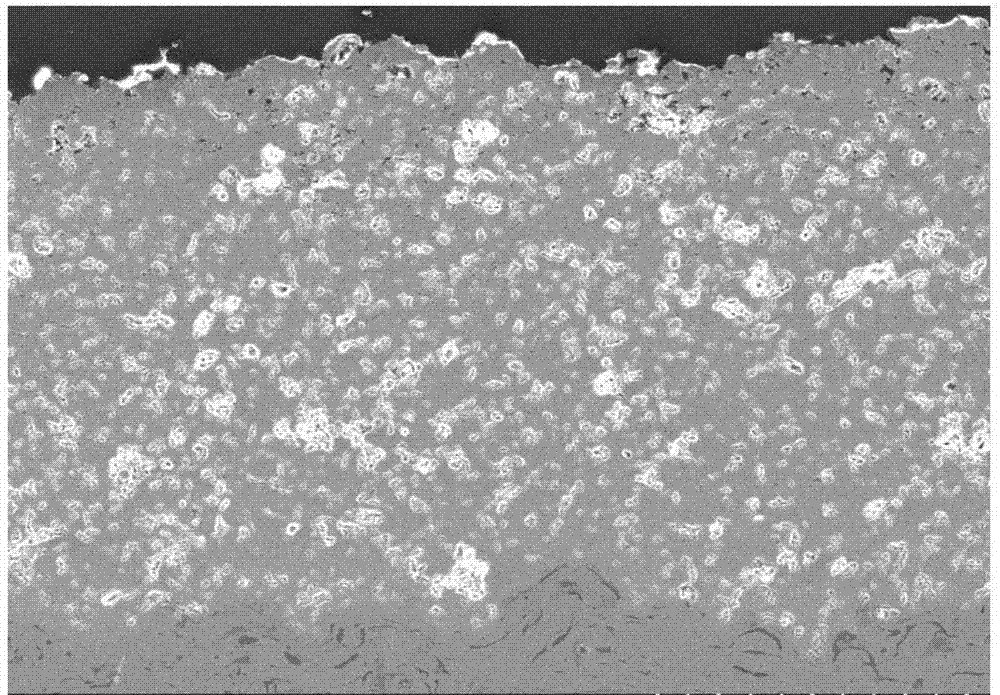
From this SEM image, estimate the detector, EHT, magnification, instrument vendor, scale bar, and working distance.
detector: SE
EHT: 20 kV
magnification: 0.2 K X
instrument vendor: Hitachi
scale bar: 200000 nm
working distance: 11.1 mm